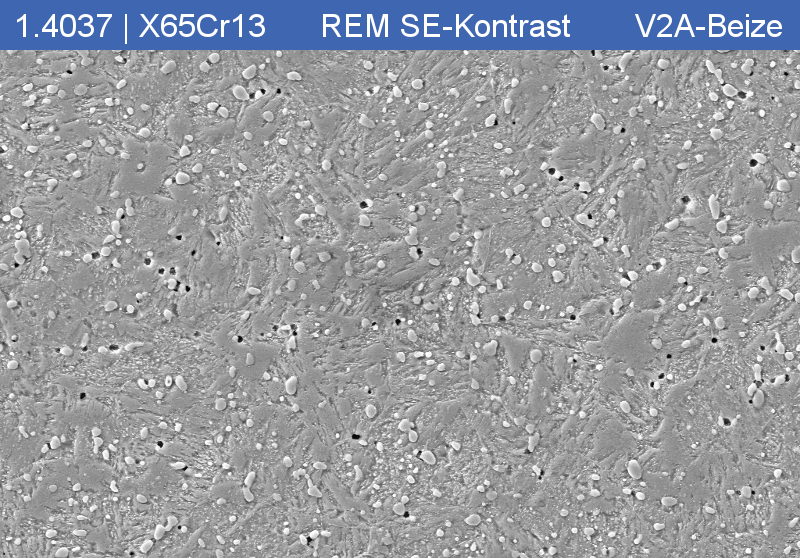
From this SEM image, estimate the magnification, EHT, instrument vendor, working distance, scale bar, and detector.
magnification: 2 K X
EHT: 15 kV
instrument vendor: Zeiss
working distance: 11 mm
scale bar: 2000 nm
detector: SE2